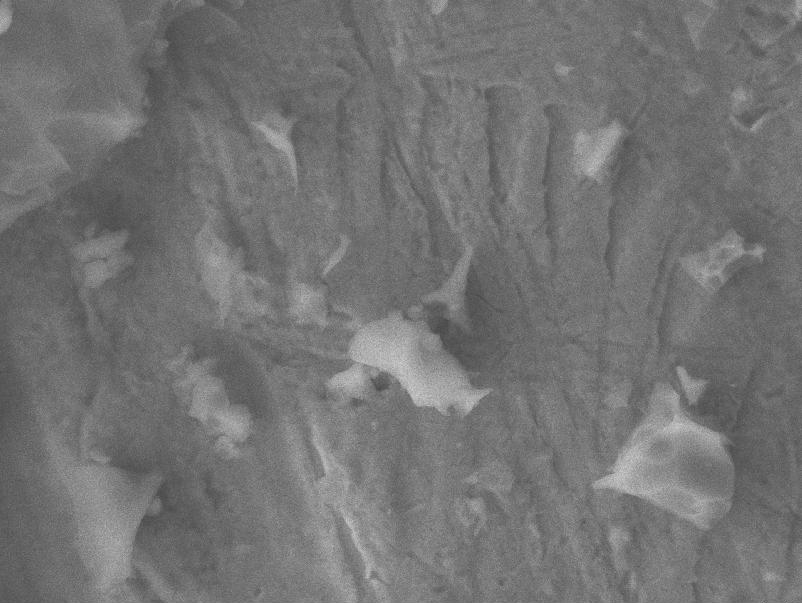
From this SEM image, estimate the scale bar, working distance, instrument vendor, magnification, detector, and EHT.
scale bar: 1000 nm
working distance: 10.7 mm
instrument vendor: JEOL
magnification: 3.3 K X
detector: LEI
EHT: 15 kV